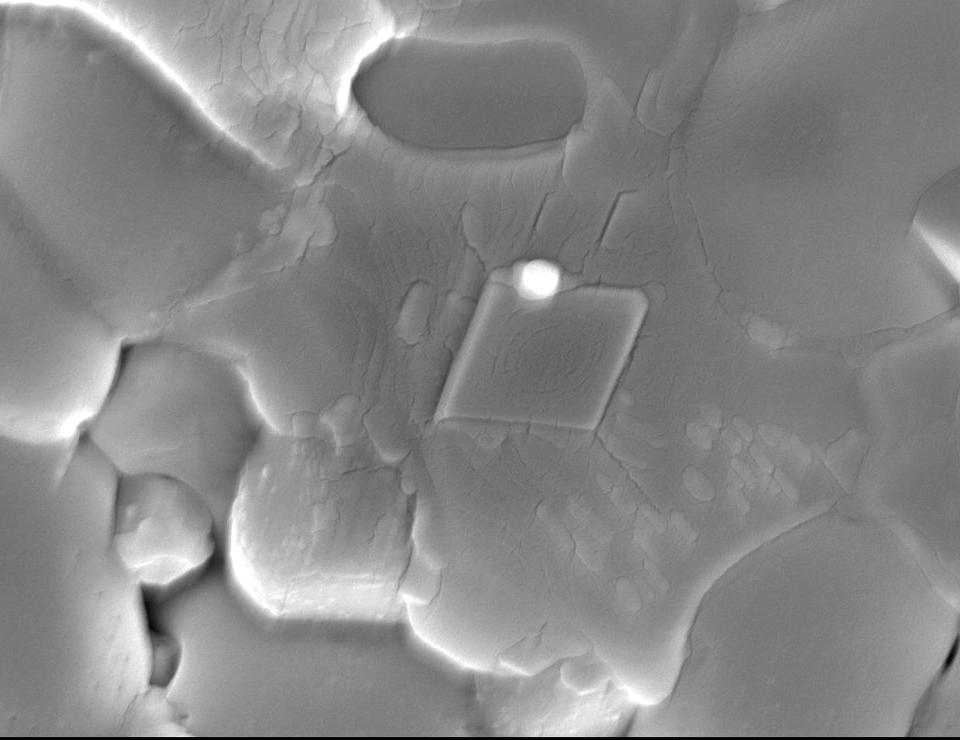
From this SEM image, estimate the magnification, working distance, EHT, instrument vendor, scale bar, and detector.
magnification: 10 K X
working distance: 13.3 mm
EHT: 15 kV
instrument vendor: JEOL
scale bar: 1000 nm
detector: SEI